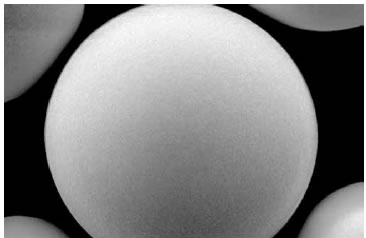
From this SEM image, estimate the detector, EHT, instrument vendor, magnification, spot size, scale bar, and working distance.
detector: SE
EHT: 5 kV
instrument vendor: FEI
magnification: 4.5 K X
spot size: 3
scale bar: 5000 nm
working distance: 11.3 mm